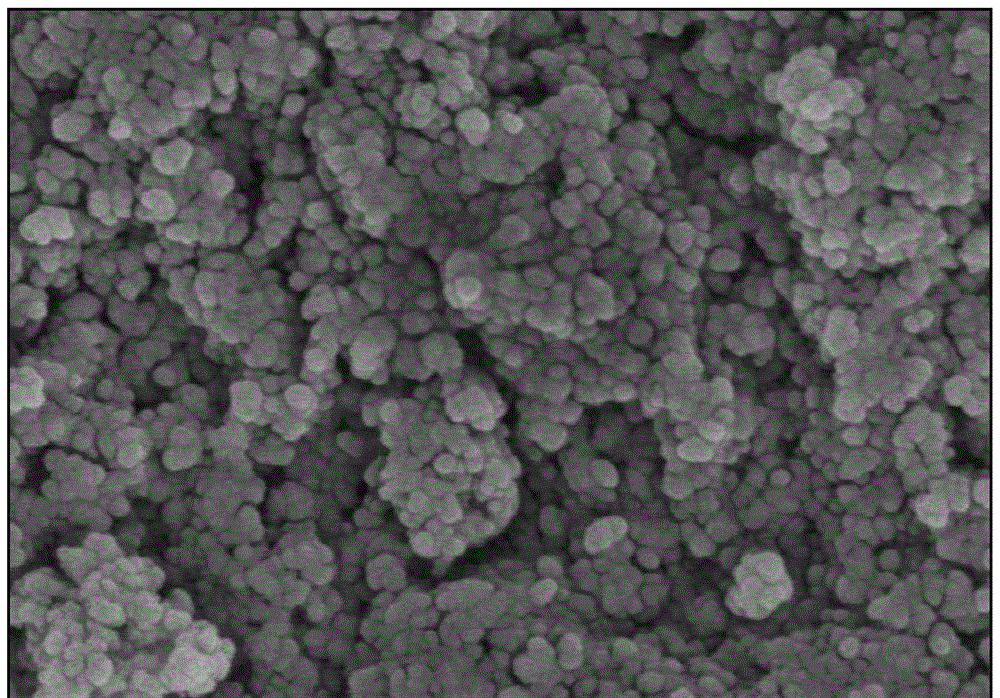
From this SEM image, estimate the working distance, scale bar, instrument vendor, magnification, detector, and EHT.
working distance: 8.7 mm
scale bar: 500 nm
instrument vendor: Hitachi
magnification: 90 K X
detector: SE(M)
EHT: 3 kV